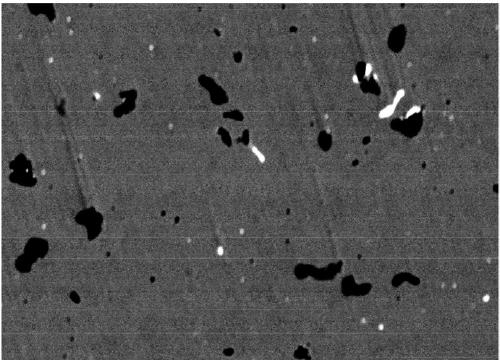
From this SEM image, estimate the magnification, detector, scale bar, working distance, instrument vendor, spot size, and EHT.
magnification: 1.2 K X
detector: BEC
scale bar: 10000 nm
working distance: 12 mm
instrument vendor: JEOL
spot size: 50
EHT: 20 kV